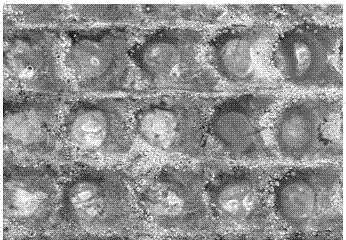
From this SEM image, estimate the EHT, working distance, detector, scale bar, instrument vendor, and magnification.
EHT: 20 kV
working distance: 13.5 mm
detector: SE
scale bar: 100000 nm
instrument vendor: Hitachi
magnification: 0.3 K X